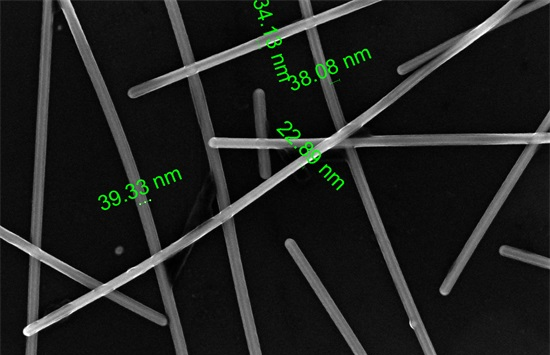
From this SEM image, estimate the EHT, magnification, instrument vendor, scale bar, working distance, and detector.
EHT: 10 kV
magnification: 200 K X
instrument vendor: FEI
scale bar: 500 nm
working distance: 4.1 mm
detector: TLD